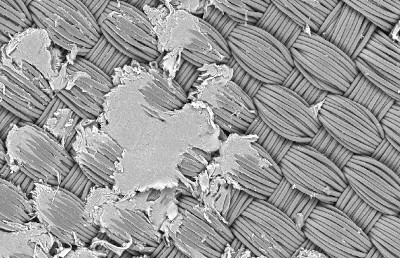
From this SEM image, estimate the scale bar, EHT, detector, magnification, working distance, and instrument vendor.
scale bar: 100000 nm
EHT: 20 kV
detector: HDBSD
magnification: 0.1 K X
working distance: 7.9 mm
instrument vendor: Zeiss